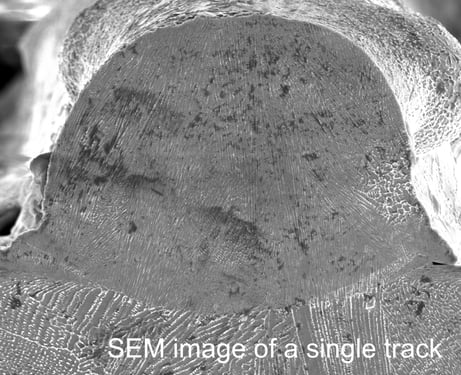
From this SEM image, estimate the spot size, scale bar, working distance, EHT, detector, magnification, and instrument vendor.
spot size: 3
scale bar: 40000 nm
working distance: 5.3 mm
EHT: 15 kV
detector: ETD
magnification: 1.578 K X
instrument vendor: FEI